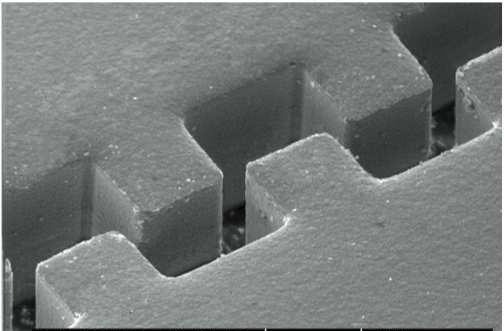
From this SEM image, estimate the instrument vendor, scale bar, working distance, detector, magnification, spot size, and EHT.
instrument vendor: FEI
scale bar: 10000 nm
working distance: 10.9 mm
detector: SE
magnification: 2.371 K X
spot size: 3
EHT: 10 kV